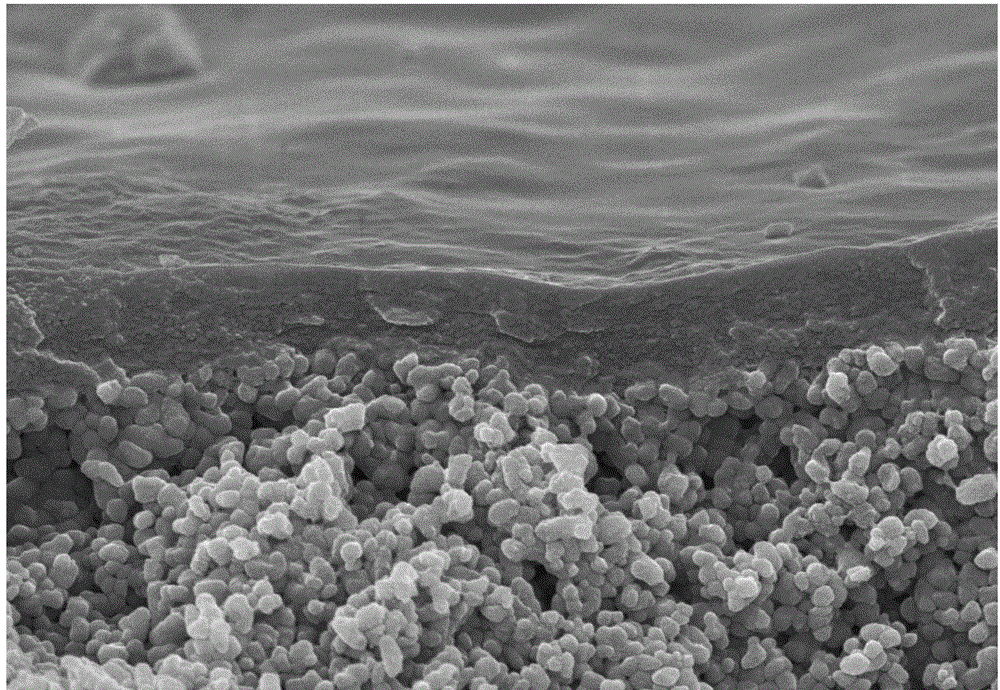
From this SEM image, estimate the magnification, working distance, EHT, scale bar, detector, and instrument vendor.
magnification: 20 K X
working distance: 8.7 mm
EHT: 10 kV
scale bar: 2000 nm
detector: SE(U)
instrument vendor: Hitachi